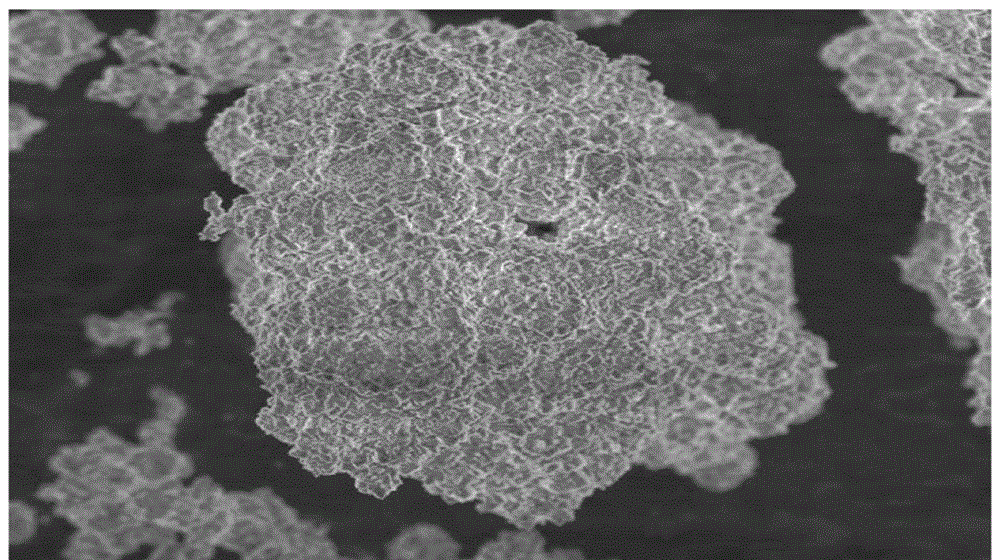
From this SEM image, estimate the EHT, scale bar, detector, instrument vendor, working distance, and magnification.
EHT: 5 kV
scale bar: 10000 nm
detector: SEI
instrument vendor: JEOL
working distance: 8 mm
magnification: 2.5 K X